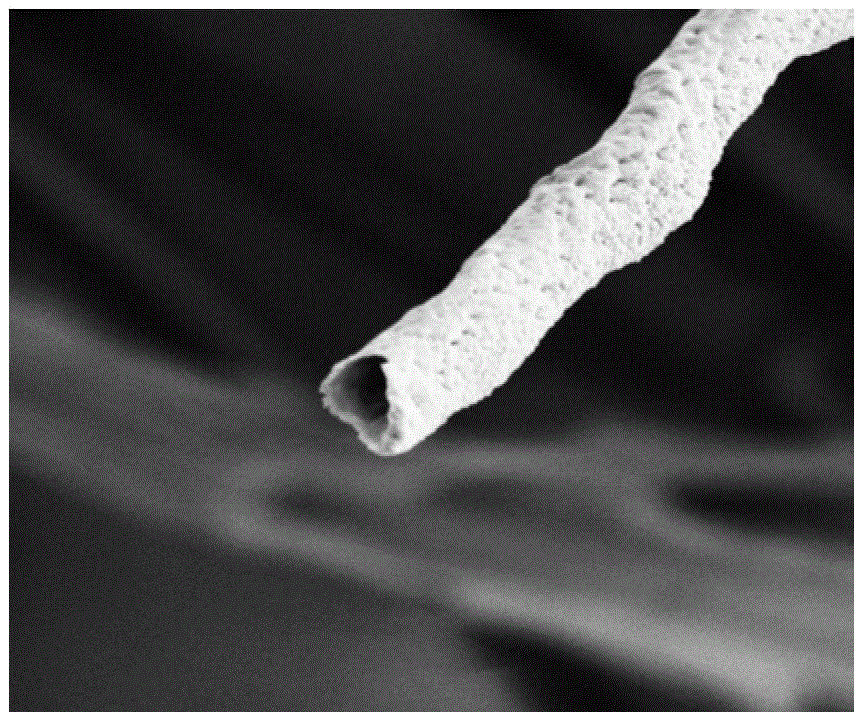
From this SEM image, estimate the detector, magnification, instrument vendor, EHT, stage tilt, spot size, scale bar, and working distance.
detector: SED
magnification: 25 K X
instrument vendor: FEI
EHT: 5 kV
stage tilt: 52°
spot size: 3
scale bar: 2000 nm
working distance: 5.026 mm